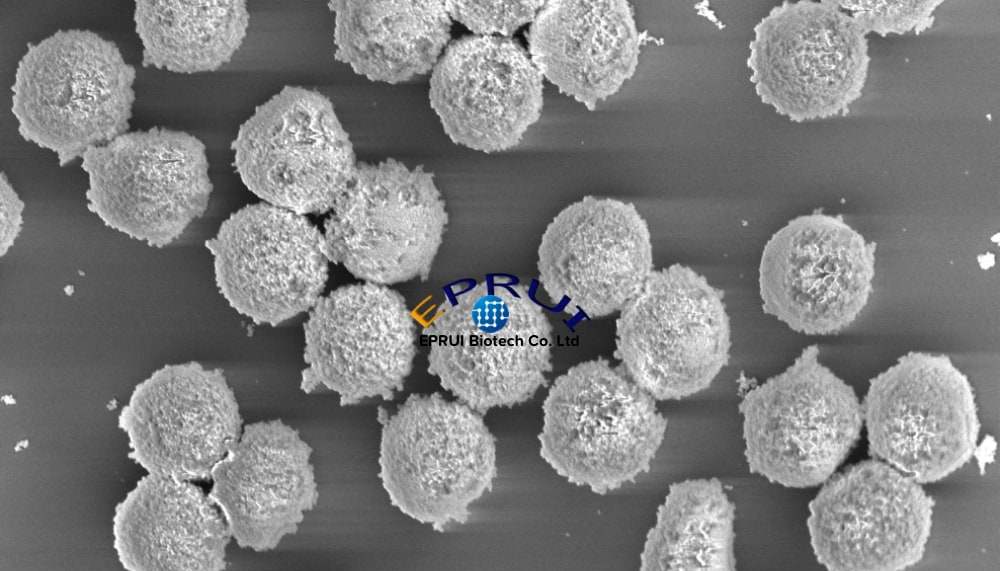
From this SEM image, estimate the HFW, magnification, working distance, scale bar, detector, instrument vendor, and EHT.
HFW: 13.8 µm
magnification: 30 K X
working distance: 5.1 mm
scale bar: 4000 nm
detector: TLD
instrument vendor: FEI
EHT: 5 kV